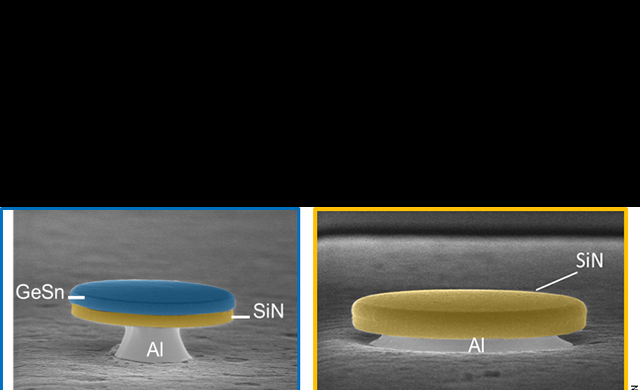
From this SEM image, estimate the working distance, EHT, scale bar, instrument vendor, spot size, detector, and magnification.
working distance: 5.2 mm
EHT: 10 kV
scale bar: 2000 nm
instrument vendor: FEI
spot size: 2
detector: TLD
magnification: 10 K X